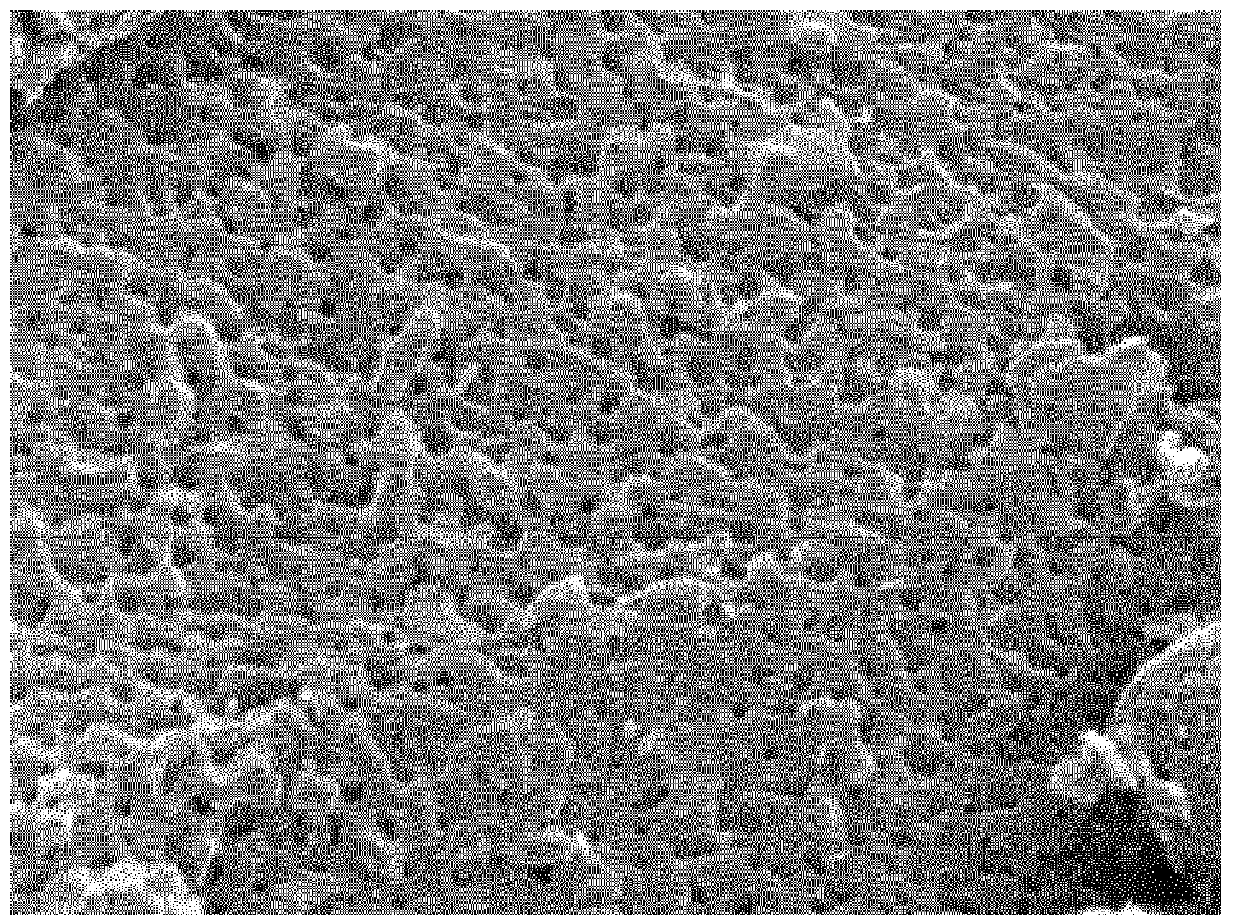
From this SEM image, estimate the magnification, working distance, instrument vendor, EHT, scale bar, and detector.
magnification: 50 K X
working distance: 2.6 mm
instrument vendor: JEOL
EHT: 0.5 kV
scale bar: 100 nm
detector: SEI